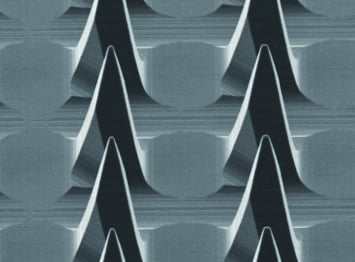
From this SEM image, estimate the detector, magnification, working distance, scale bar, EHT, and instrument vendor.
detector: SEI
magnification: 0.095 K X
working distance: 25 mm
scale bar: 100000 nm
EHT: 15 kV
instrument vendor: JEOL